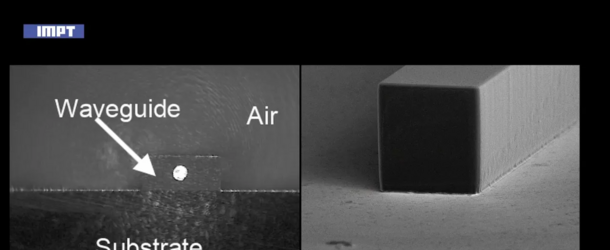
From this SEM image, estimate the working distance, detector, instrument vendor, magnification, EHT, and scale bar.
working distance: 37 mm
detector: SE1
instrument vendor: Zeiss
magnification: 0.258 K X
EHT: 15 kV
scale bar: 20000 nm